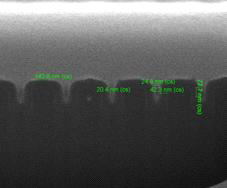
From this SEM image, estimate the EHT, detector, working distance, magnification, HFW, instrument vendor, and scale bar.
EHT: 5 kV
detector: ETD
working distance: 9.9 mm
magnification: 200 K X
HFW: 1.49 µm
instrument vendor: FEI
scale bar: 500 nm